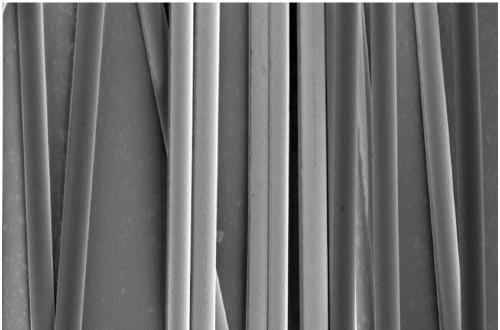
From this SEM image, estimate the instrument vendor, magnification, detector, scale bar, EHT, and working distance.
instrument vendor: Zeiss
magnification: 1 K X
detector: InLens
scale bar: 10000 nm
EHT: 1 kV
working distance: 4.7 mm